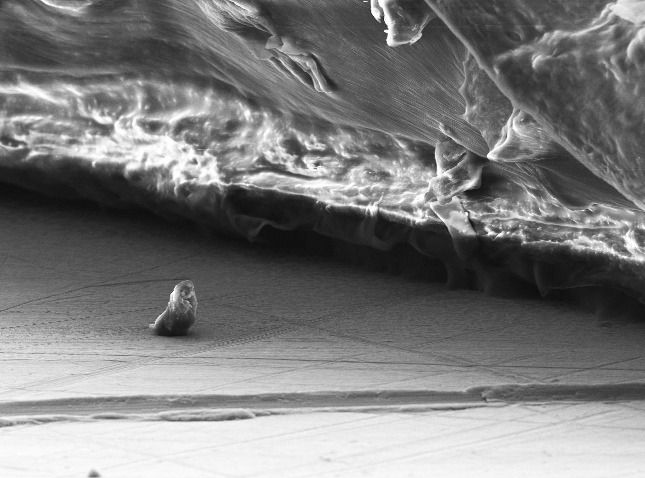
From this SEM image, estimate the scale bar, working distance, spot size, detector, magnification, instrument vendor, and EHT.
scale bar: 20000 nm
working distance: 13.2 mm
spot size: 3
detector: SE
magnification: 1.084 K X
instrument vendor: FEI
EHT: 10 kV